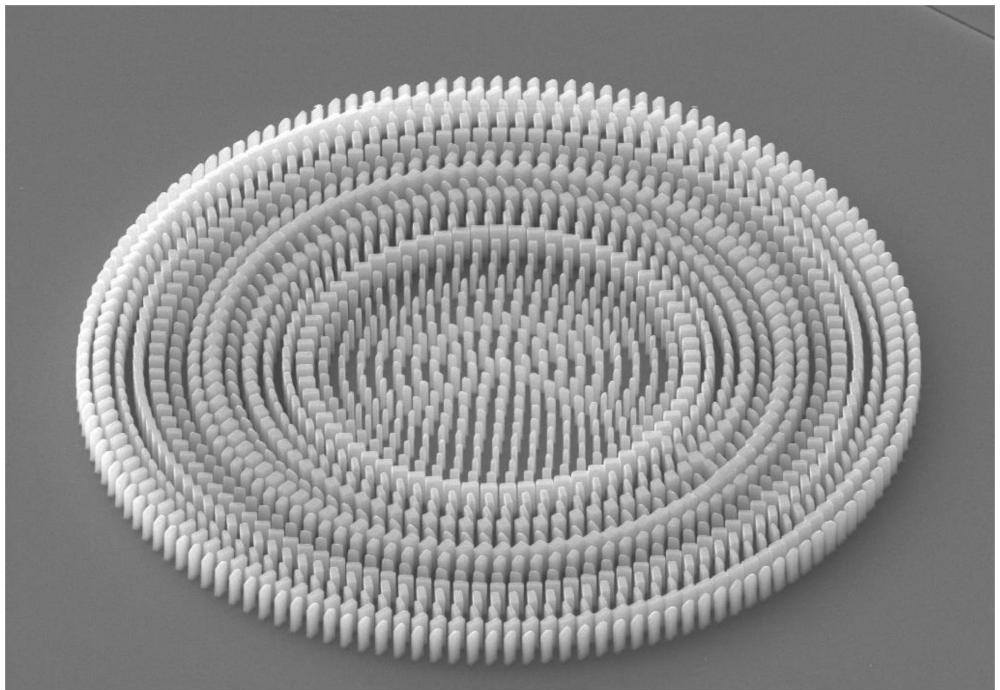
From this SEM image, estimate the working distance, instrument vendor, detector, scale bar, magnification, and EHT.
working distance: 14.4 mm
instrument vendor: Zeiss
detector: SE2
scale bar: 1000 nm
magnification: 5.26 K X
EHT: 10 kV